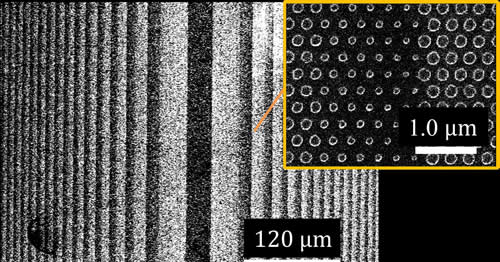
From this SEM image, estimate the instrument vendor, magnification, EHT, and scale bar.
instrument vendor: JEOL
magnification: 0.25 K X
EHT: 10 kV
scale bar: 120000 nm